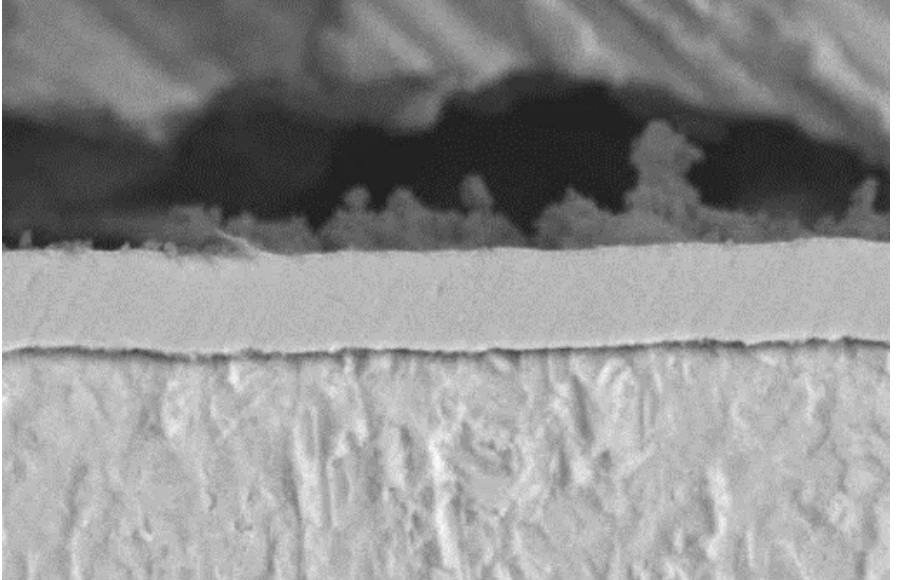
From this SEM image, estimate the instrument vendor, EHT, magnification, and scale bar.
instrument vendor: JEOL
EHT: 20 kV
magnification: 3 K X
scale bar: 5000 nm